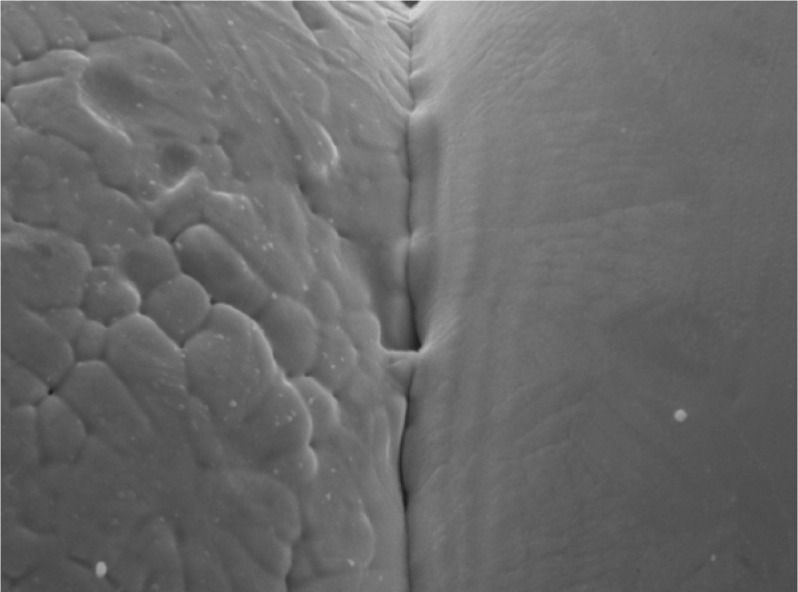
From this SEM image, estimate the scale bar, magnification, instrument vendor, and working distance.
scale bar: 1000 nm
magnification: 5.5 K X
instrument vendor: JEOL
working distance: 38 mm